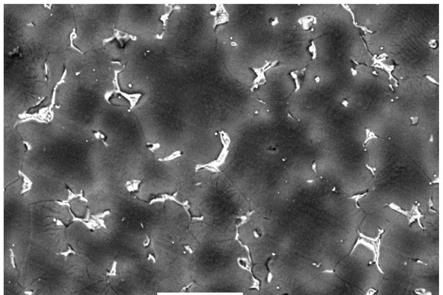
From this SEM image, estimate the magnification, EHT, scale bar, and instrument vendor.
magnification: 0.5 K X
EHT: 20 kV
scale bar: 50000 nm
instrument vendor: JEOL